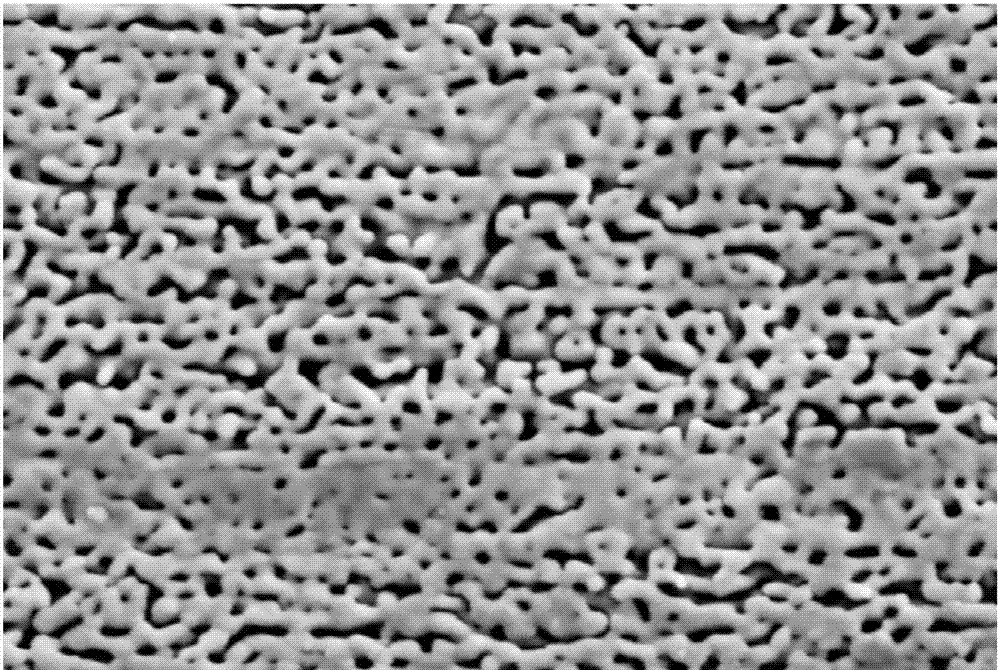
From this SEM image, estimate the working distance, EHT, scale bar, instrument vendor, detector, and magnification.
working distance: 7.9 mm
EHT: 5 kV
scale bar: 100 nm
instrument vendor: Zeiss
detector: SE2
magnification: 100 K X